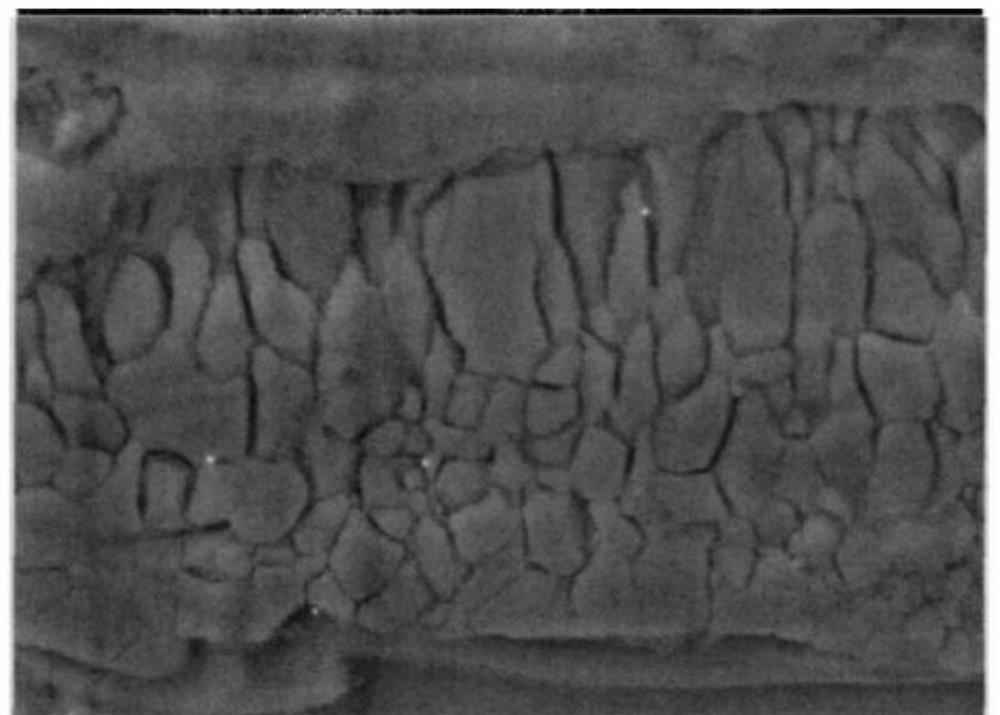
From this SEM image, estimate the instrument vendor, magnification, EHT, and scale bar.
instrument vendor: other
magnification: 3 K X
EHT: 30 kV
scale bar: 10000 nm